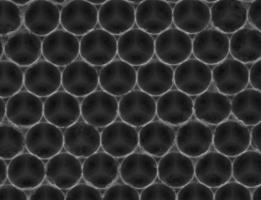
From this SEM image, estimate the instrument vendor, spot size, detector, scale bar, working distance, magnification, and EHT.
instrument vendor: FEI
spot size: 3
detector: SE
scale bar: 1000 nm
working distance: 10 mm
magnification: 100 K X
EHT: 10 kV